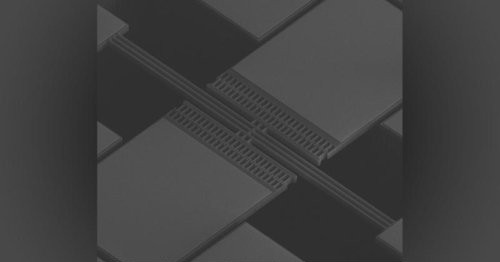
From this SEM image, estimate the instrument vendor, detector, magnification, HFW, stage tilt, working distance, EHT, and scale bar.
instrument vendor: FEI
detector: ETD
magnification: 0.325 K X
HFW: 394 µm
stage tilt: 45°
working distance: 5.4 mm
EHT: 15 kV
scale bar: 100000 nm